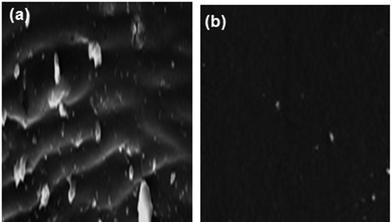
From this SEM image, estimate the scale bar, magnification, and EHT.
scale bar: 20000 nm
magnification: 3 K X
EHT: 30 kV